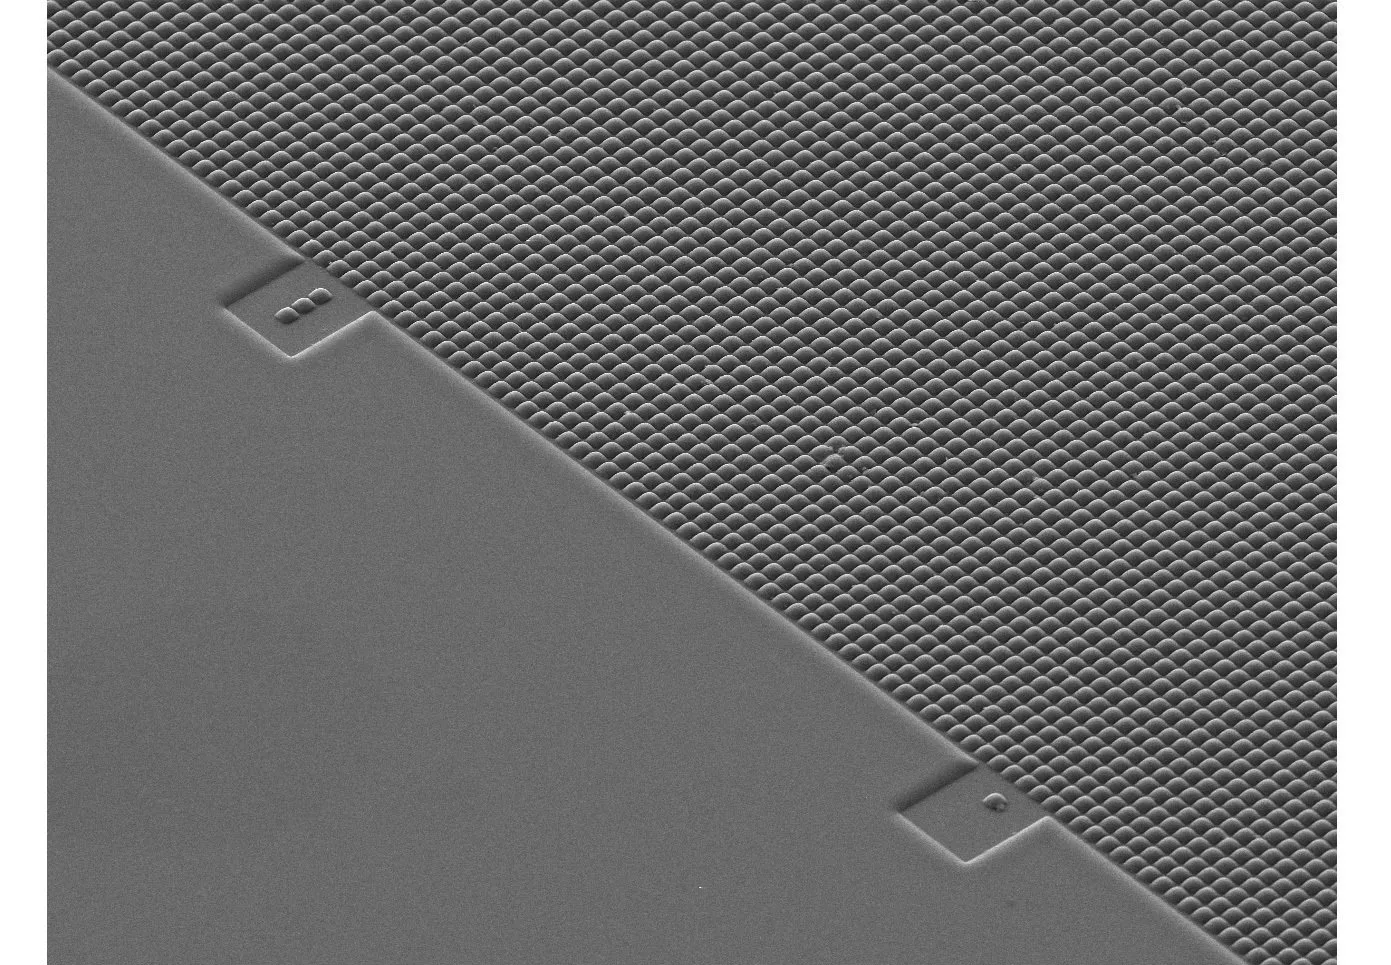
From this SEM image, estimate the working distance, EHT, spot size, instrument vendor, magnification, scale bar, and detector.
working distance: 6.2 mm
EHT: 10 kV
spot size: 3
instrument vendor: FEI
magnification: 0.35 K X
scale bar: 50000 nm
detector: SE